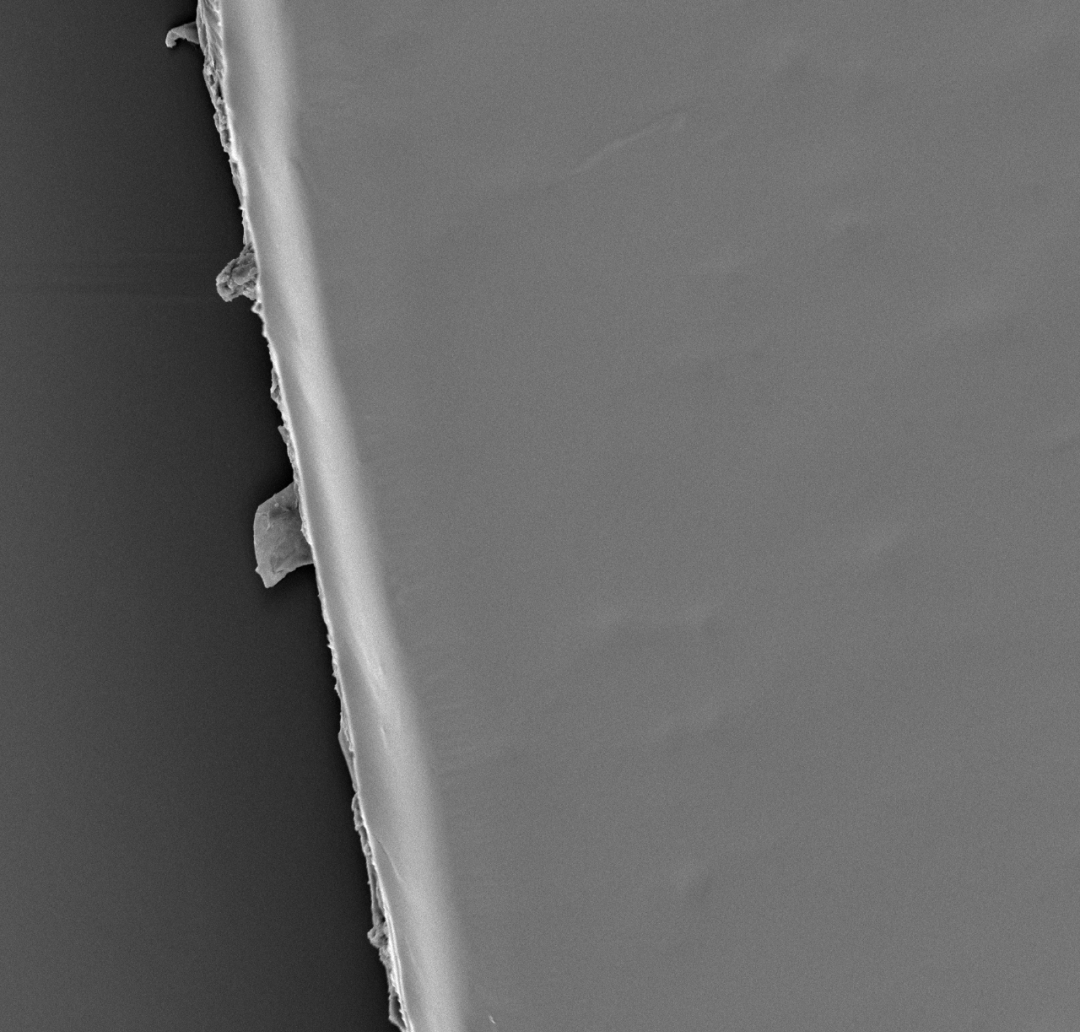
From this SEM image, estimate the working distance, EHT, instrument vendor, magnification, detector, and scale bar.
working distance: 9.1 mm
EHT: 10 kV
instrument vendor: other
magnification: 1 K X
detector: Mix 50%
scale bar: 50000 nm